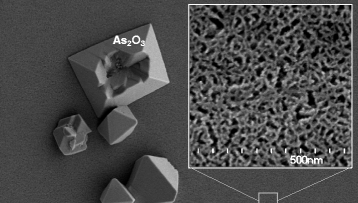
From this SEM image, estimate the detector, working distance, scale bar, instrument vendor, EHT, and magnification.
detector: SE(L)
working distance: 12.1 mm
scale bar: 5000 nm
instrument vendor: Hitachi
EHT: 3 kV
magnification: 7 K X